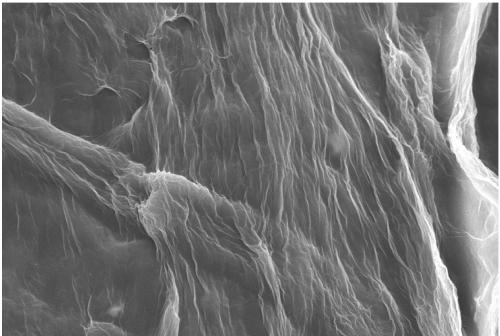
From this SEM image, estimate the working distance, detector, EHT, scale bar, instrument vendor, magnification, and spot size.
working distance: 17 mm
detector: SEI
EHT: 15 kV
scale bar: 10000 nm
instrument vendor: JEOL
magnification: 2.5 K X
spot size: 40